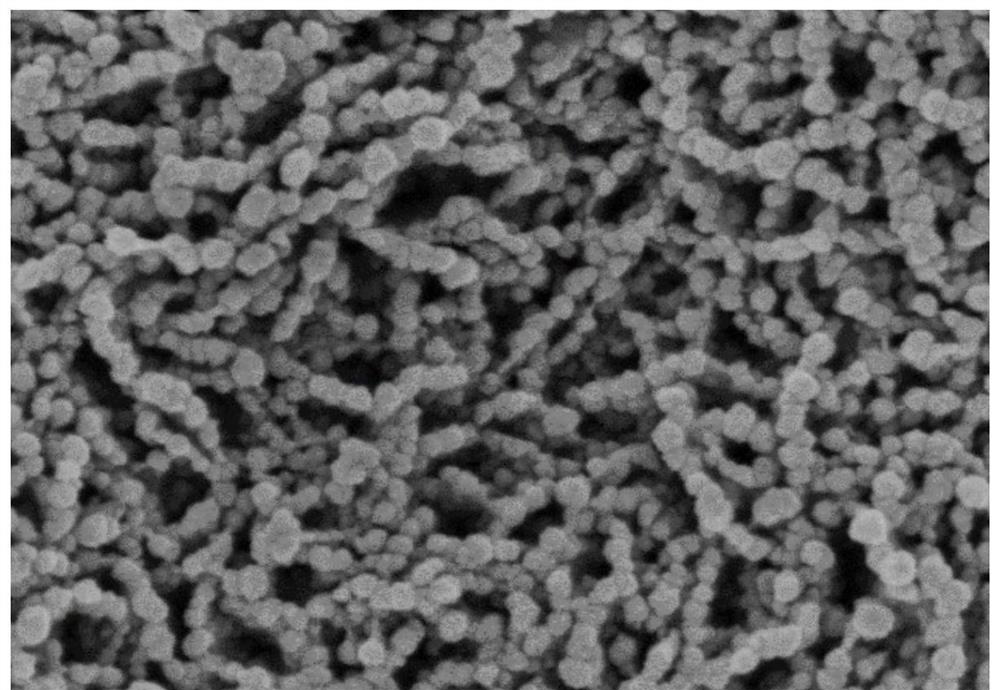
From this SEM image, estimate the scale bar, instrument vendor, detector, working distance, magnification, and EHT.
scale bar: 500 nm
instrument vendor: Hitachi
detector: SE(M)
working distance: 4.6 mm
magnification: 100 K X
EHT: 2 kV